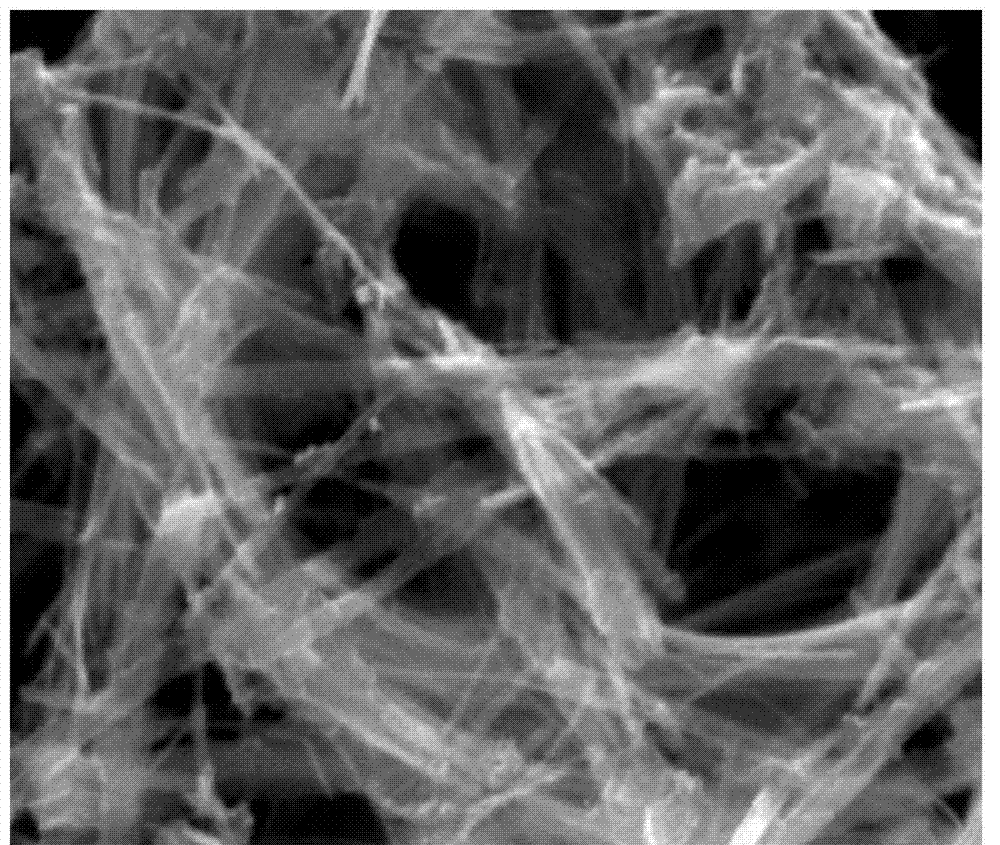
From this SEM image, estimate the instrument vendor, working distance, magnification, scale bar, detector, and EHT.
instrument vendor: FEI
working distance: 14.2 mm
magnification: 20 K X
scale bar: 3000 nm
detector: ETD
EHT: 30 kV